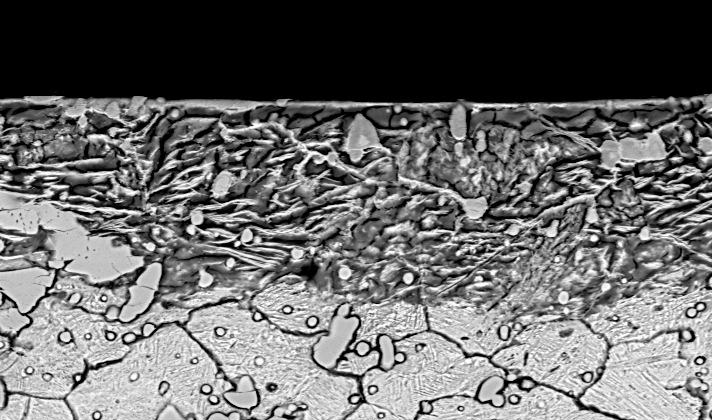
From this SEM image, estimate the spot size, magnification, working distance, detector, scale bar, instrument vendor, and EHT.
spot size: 5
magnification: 1 K X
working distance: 12.7 mm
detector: BSE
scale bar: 20000 nm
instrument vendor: FEI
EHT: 20 kV